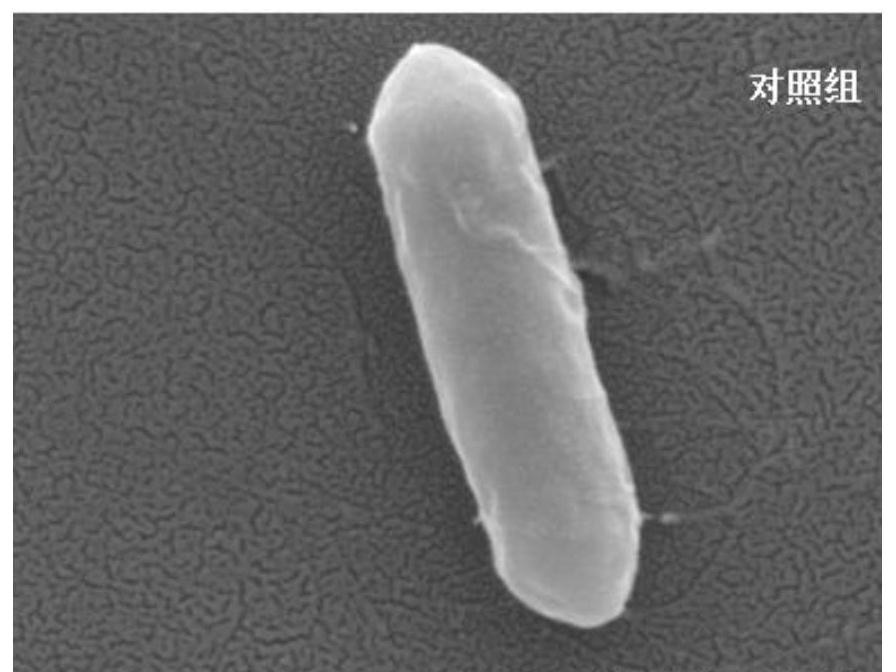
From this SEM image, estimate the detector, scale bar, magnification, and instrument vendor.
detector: LED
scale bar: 100 nm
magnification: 50 K X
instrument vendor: JEOL